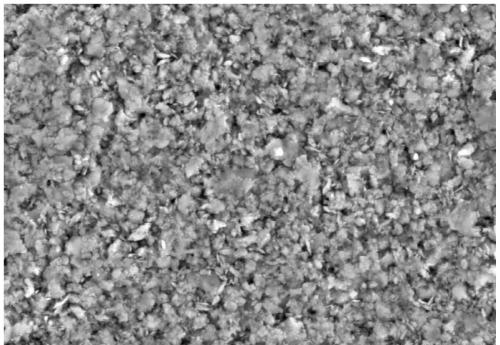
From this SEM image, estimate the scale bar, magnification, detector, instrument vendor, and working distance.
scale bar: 10000 nm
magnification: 5 K X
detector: SE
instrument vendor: Hitachi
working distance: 8.5 mm